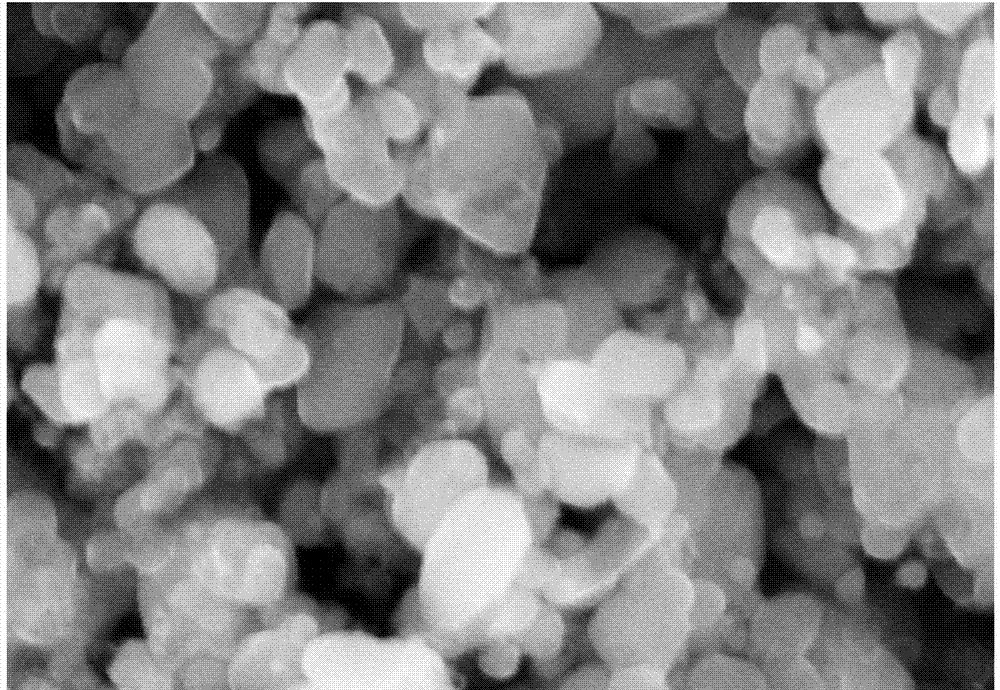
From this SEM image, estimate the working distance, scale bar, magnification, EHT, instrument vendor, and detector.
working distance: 16.3 mm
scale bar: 1000 nm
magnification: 50 K X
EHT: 15 kV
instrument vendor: Hitachi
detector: SE(M)